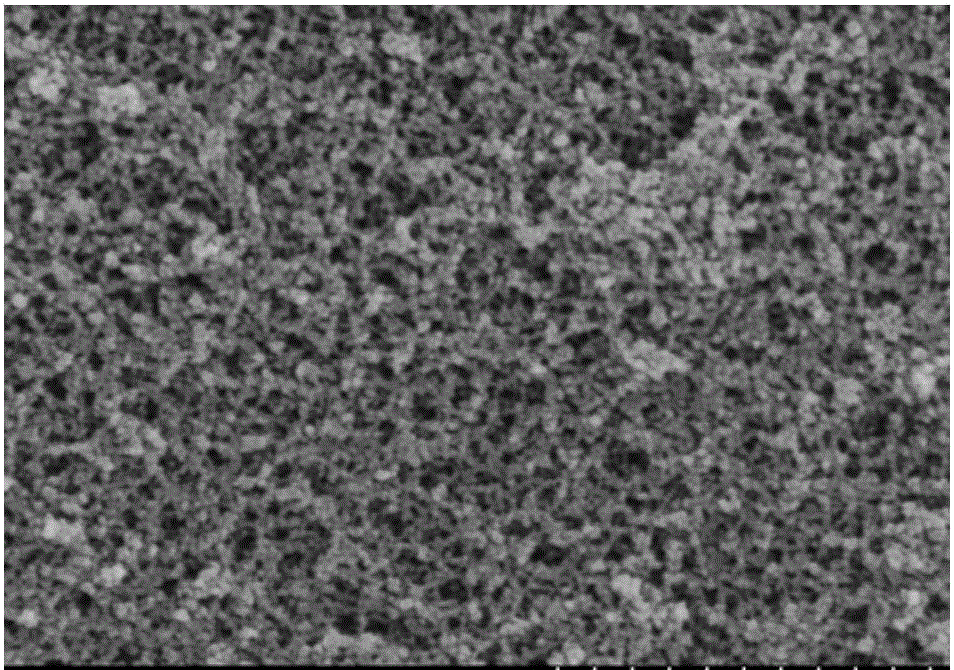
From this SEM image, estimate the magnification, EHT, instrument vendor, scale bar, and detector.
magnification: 50 K X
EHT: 4 kV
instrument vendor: Hitachi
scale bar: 1000 nm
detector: SE(M)